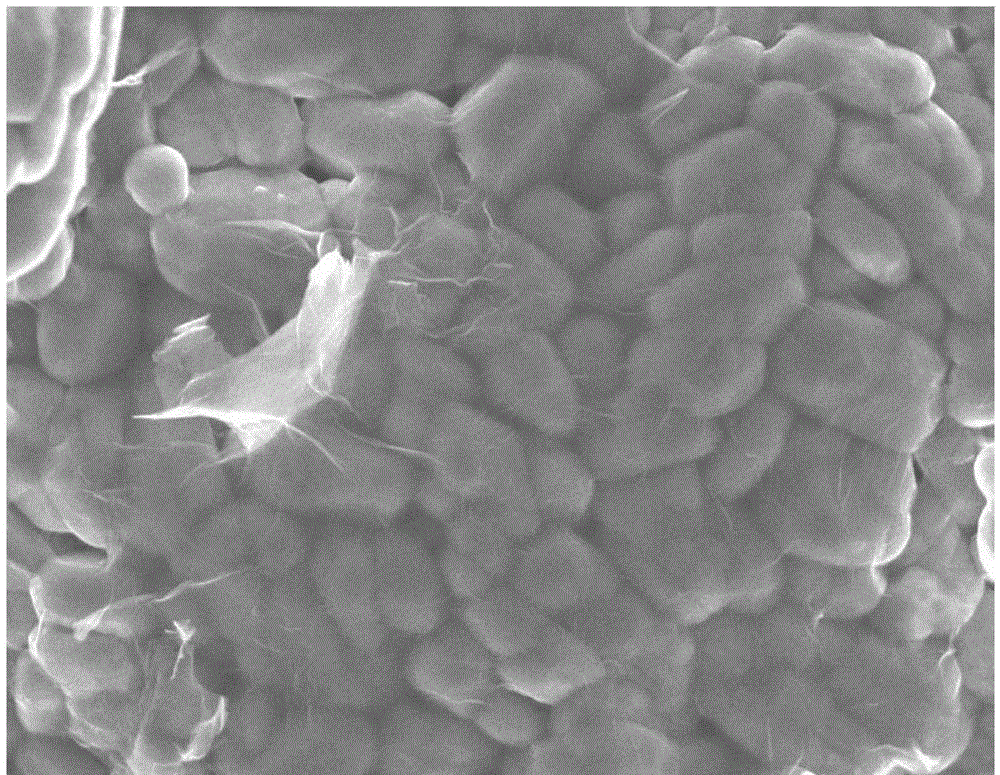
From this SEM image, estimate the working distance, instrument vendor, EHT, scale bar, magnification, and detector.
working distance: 7.8 mm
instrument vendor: JEOL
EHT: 5 kV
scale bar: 1000 nm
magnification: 20 K X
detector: SEI